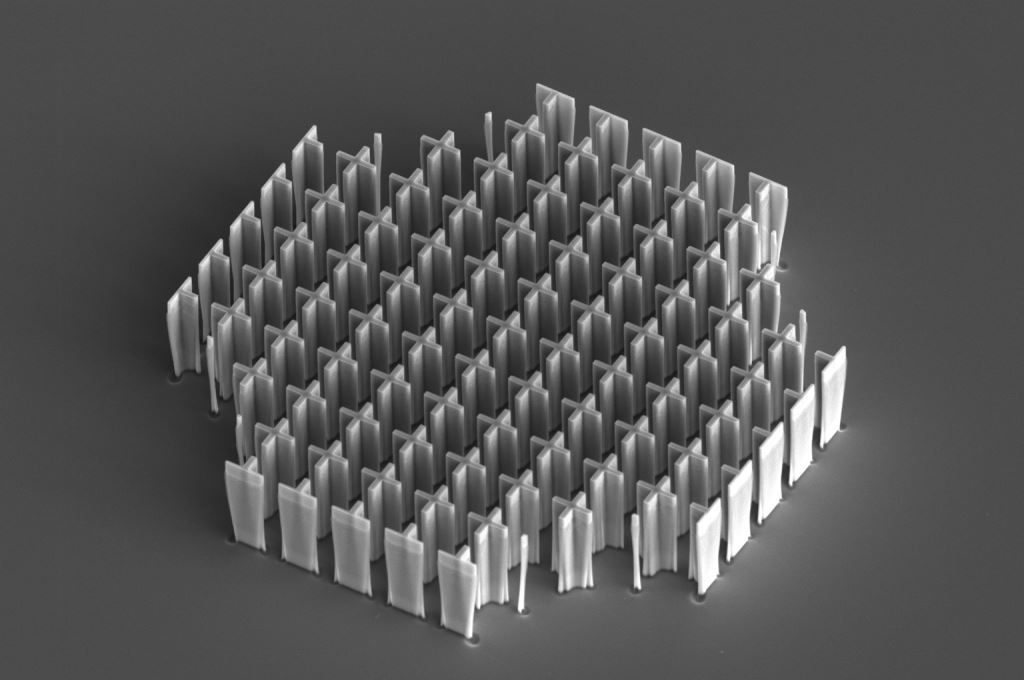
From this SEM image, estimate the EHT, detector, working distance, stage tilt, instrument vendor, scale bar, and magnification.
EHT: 10 kV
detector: ETD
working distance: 23.2 mm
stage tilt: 44.1°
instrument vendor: FEI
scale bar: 40000 nm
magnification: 2.5 K X